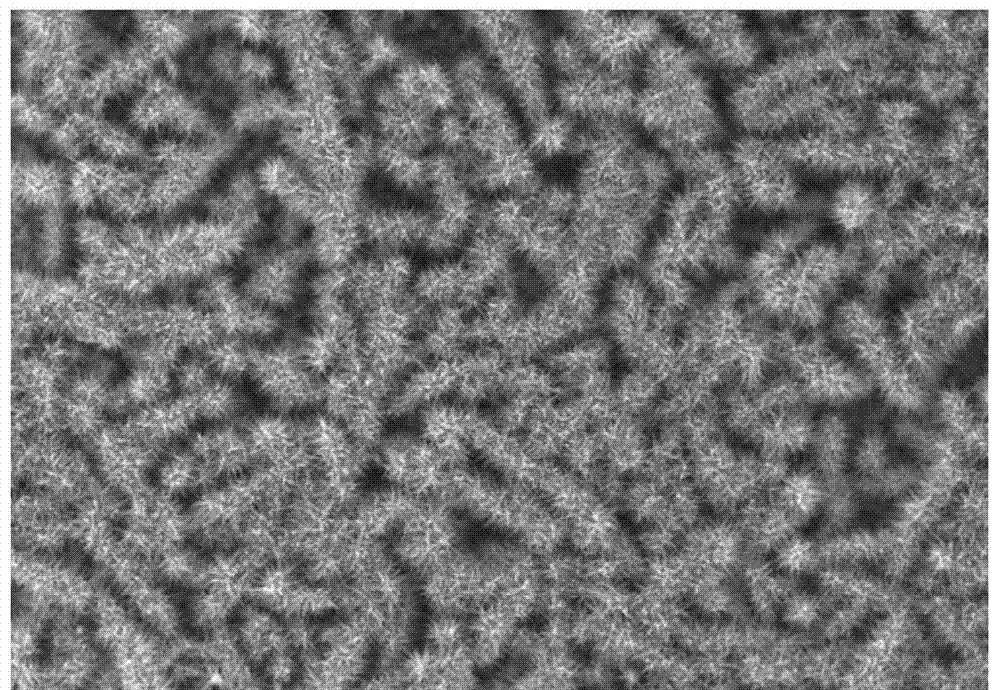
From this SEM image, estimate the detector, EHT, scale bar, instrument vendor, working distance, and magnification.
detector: SE(M)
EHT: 10 kV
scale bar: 20000 nm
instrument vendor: Hitachi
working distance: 5 mm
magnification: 2 K X